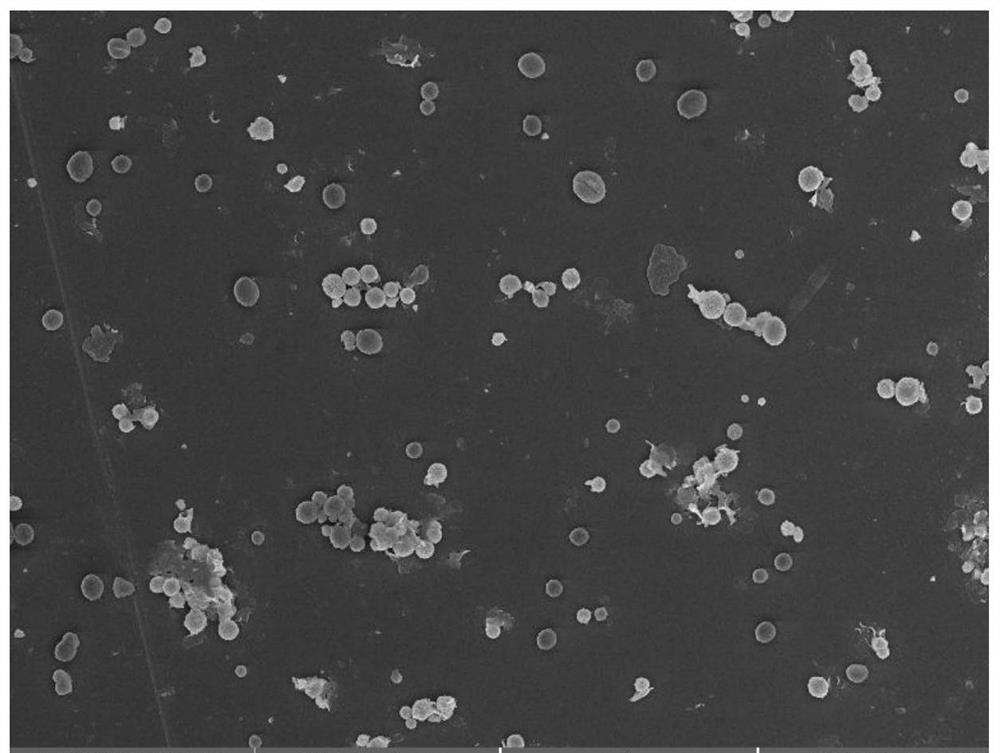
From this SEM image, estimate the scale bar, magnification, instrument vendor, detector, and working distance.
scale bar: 10000 nm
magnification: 5 K X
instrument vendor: Tescan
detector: InBeam SE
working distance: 7.28 mm